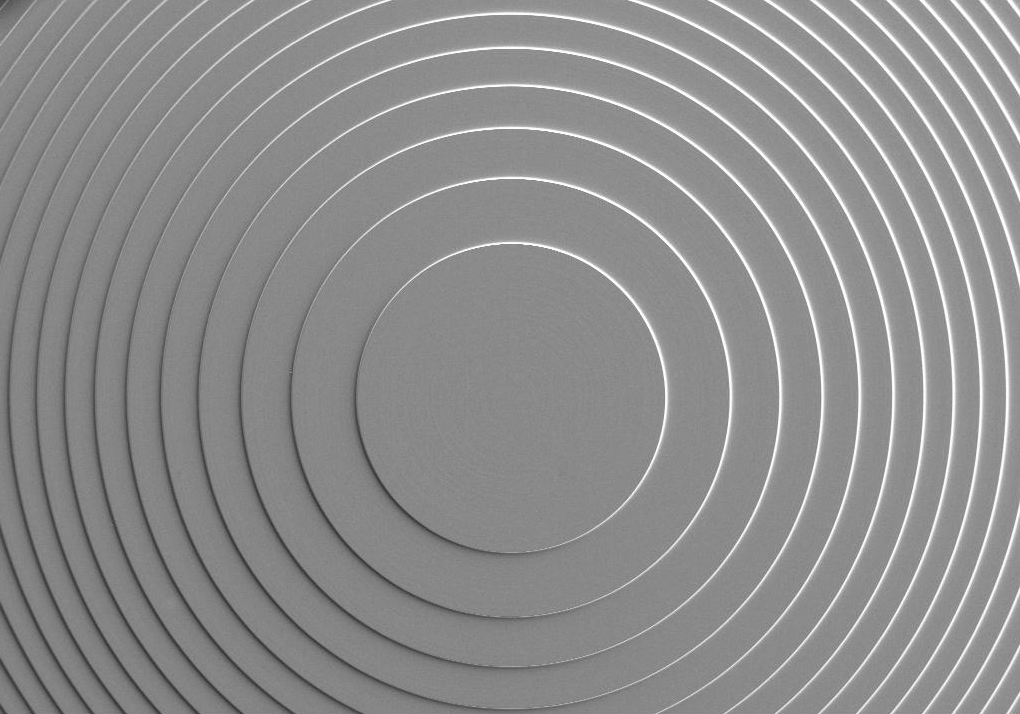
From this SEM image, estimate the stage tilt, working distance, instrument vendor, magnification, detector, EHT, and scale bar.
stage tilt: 0°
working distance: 15.4 mm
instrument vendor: Zeiss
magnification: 0.035 K X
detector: SE2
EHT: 5 kV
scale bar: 100000 nm